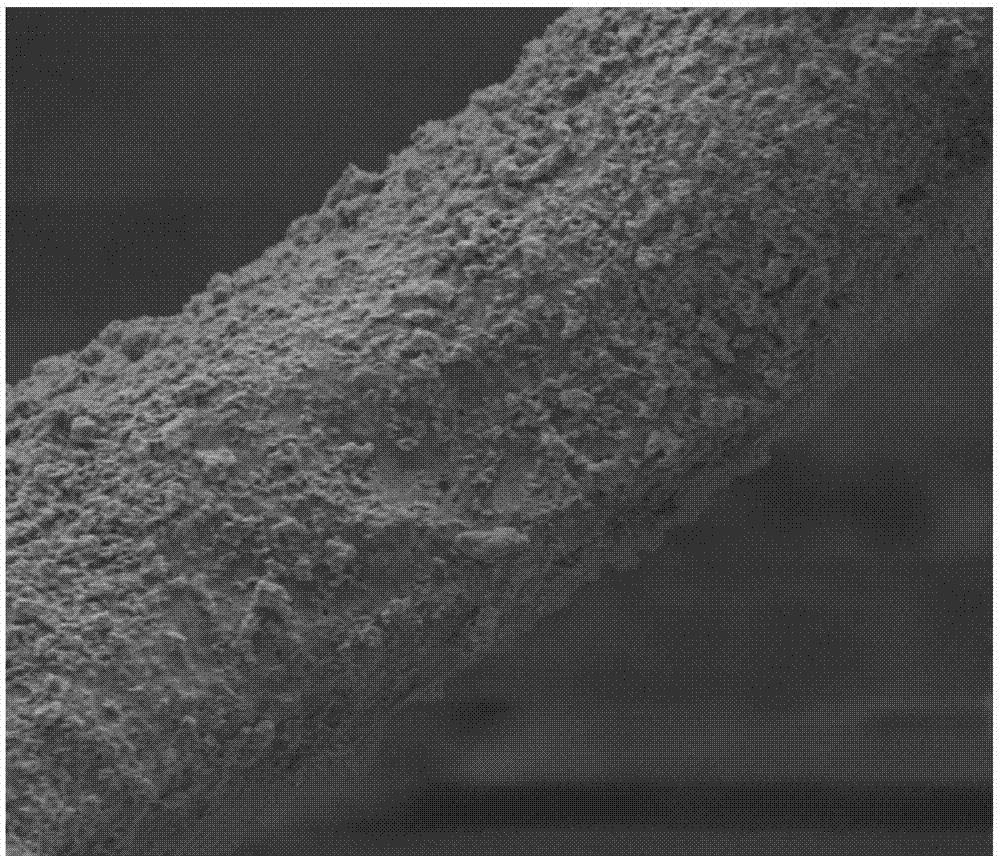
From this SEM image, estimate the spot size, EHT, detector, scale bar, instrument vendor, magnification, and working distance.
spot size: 5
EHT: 8 kV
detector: ETD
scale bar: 50000 nm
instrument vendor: FEI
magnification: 1.6 K X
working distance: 11.3 mm